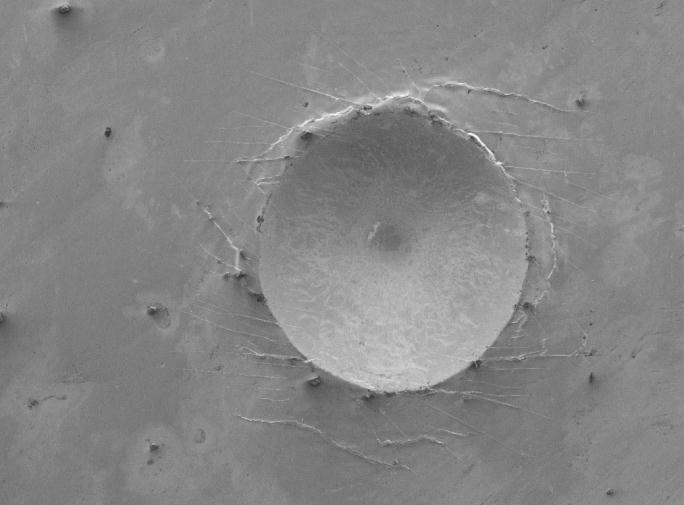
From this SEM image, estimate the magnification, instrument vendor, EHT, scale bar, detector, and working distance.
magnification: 0.05 K X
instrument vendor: JEOL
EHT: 5 kV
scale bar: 100000 nm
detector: SEI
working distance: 8 mm